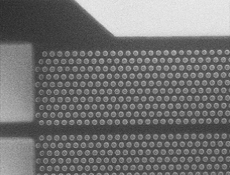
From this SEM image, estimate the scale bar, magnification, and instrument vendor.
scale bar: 2000 nm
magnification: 16 K X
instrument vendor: JEOL